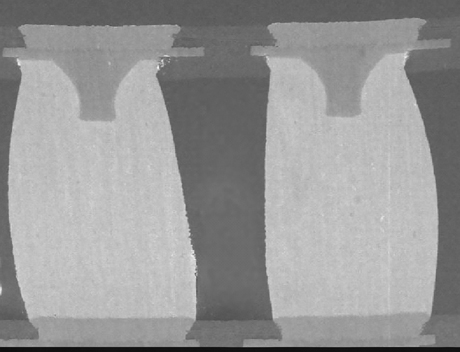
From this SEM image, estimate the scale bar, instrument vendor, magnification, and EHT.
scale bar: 200000 nm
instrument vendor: JEOL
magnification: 0.05 K X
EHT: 15 kV